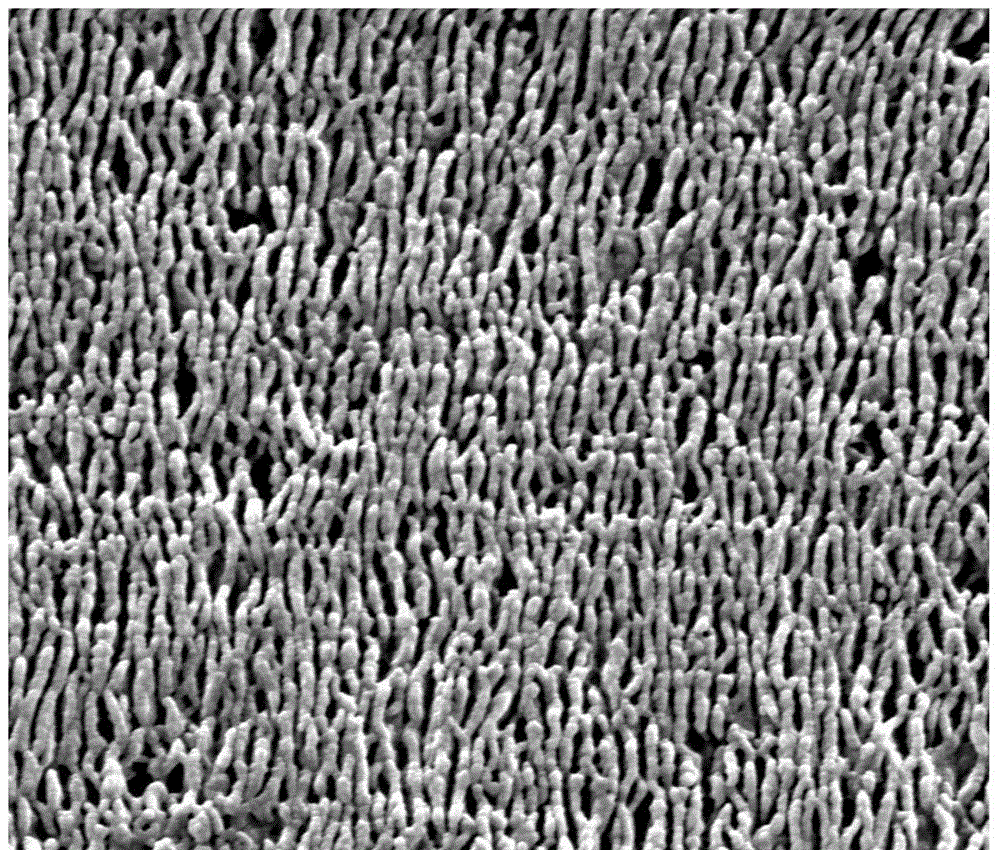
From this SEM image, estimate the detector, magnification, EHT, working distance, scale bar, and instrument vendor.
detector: SE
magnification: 80 K X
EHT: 20 kV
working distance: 10.6 mm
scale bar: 1000 nm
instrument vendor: FEI